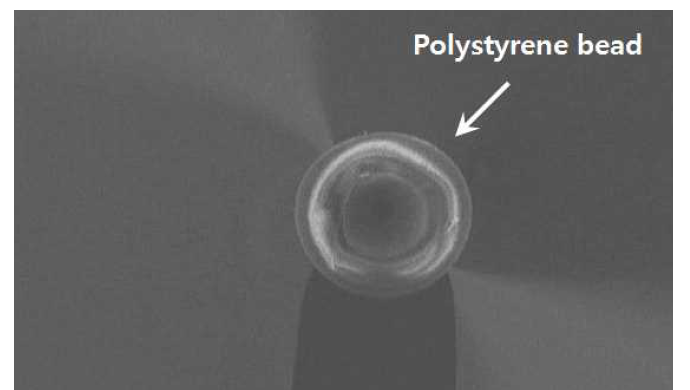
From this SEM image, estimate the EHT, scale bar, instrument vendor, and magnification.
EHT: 5 kV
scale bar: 10000 nm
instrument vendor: Hitachi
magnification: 0.75 K X